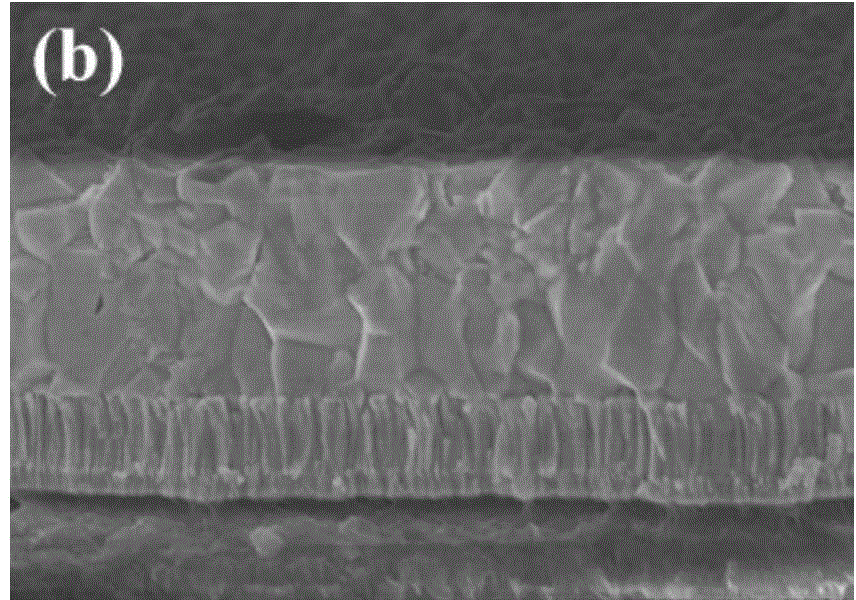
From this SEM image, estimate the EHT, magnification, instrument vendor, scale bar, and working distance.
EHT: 3 kV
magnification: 30 K X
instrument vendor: Hitachi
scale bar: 1000 nm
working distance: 9 mm